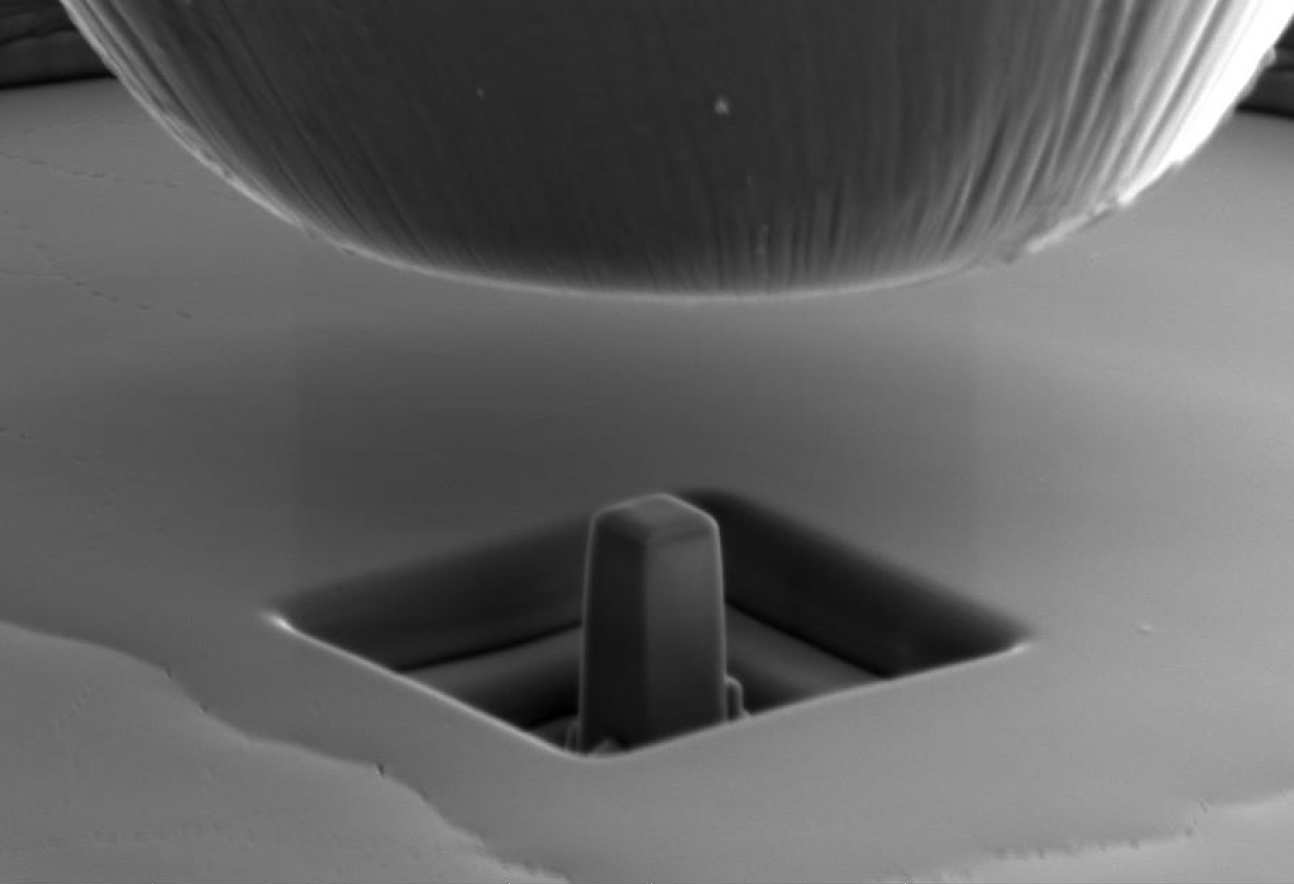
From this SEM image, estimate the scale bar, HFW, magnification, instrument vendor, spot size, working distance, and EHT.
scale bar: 3000 nm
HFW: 16.5 µm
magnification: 25.051 K X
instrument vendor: FEI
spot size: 4.5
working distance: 13.8 mm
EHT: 5 kV